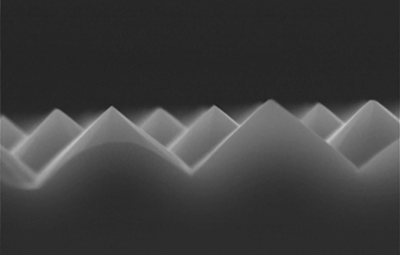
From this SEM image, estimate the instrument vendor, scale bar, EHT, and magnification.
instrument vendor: JEOL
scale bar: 1000 nm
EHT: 15 kV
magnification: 10 K X